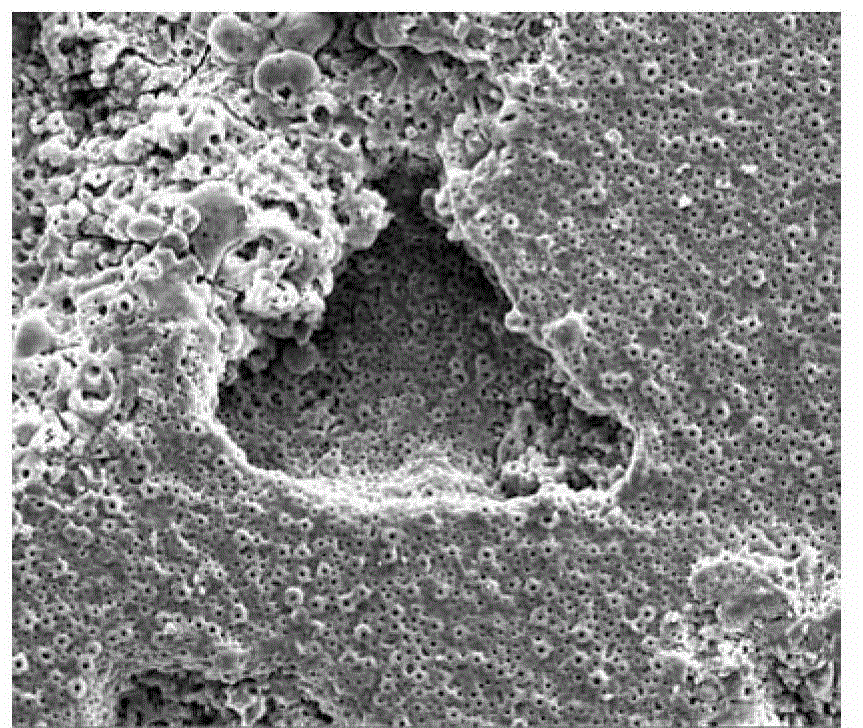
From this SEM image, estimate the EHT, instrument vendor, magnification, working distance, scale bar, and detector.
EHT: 20 kV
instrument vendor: FEI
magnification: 1 K X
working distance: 5.9 mm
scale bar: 50000 nm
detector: SE